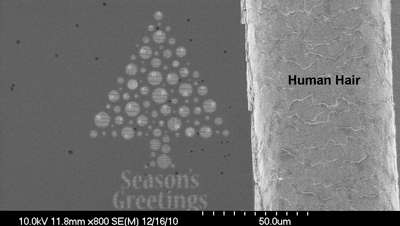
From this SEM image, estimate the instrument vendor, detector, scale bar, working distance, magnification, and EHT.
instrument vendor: Hitachi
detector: SE(M)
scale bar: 50000 nm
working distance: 11.8 mm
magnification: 0.8 K X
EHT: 10 kV